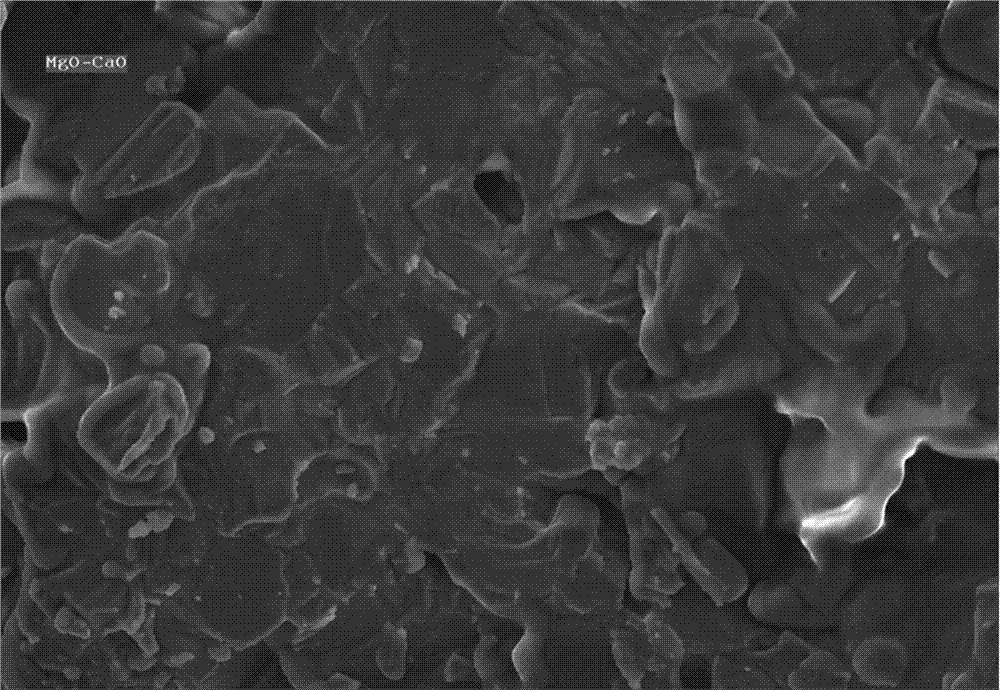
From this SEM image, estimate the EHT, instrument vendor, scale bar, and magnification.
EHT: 20 kV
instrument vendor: other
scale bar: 100000 nm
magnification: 0.4 K X